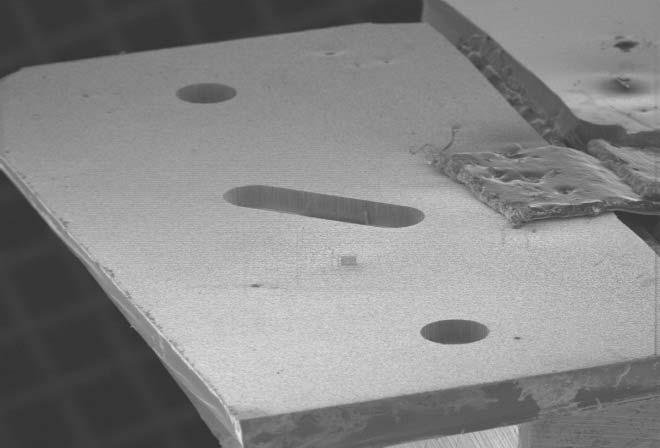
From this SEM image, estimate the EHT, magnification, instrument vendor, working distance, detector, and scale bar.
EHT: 5 kV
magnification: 0.015 K X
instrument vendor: JEOL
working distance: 48 mm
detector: SEI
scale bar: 1000000 nm